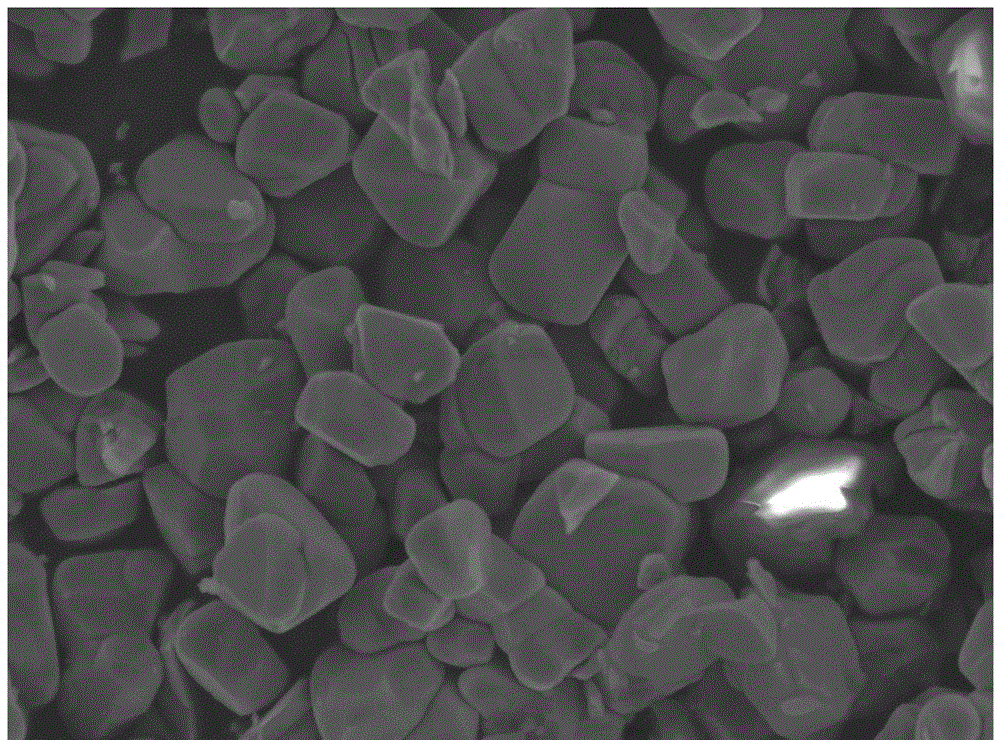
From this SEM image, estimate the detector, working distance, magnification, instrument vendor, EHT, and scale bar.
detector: LED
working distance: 9.6 mm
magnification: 10 K X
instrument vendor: JEOL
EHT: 5 kV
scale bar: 1000 nm